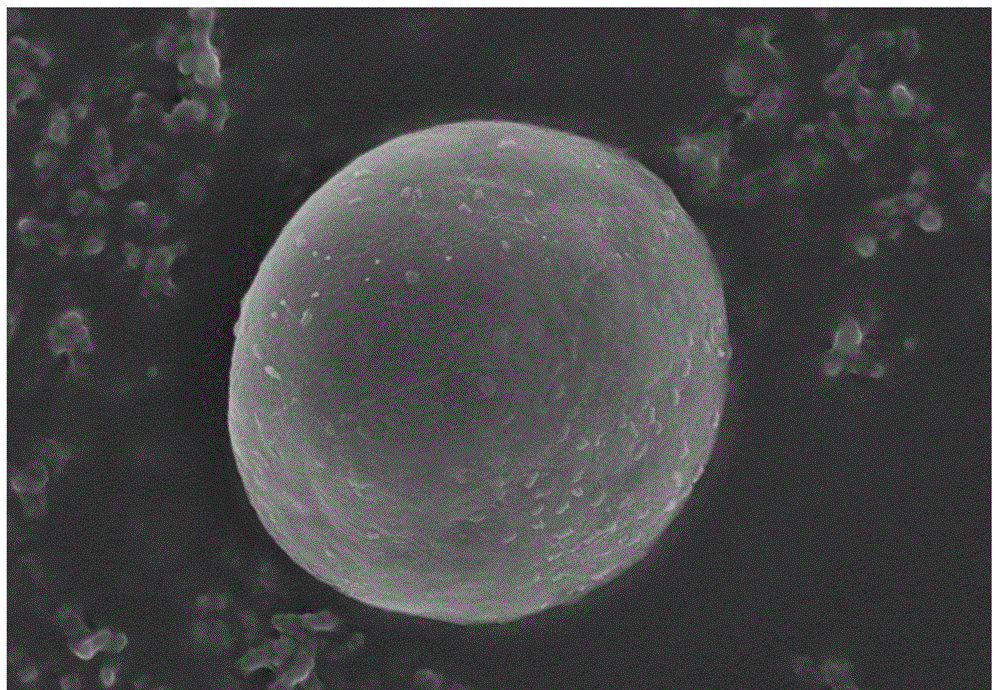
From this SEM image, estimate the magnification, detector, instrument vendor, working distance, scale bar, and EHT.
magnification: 25 K X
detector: SE(M,LA0)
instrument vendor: Hitachi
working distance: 8.4 mm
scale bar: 2000 nm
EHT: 5 kV